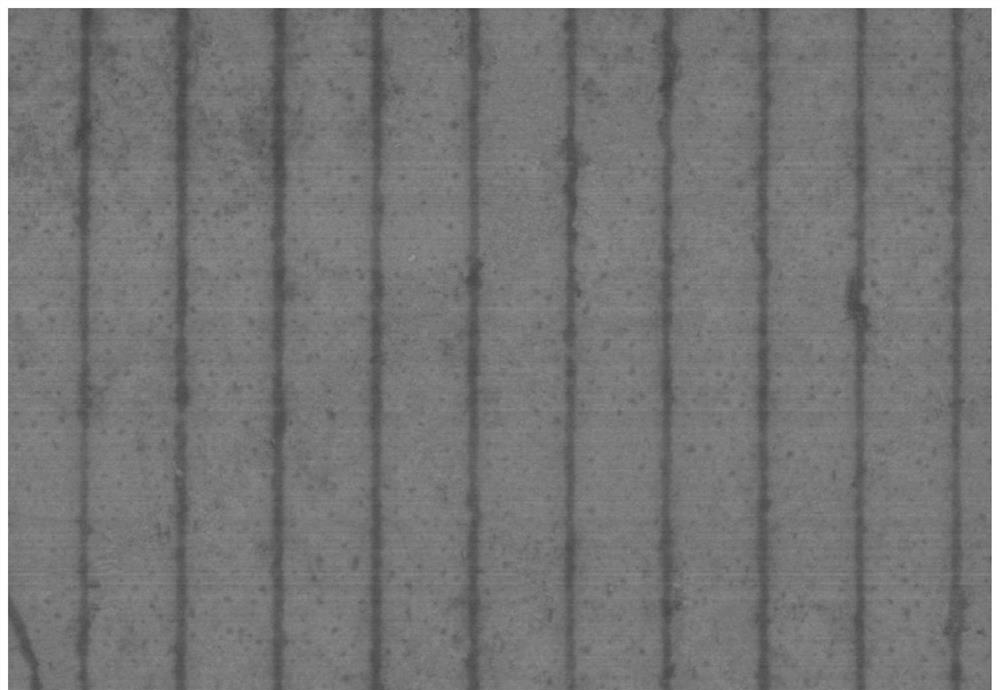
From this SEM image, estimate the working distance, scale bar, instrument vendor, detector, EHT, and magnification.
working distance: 9.1 mm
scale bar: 2000 nm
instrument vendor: Hitachi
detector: SE(M)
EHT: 3 kV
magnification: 20 K X